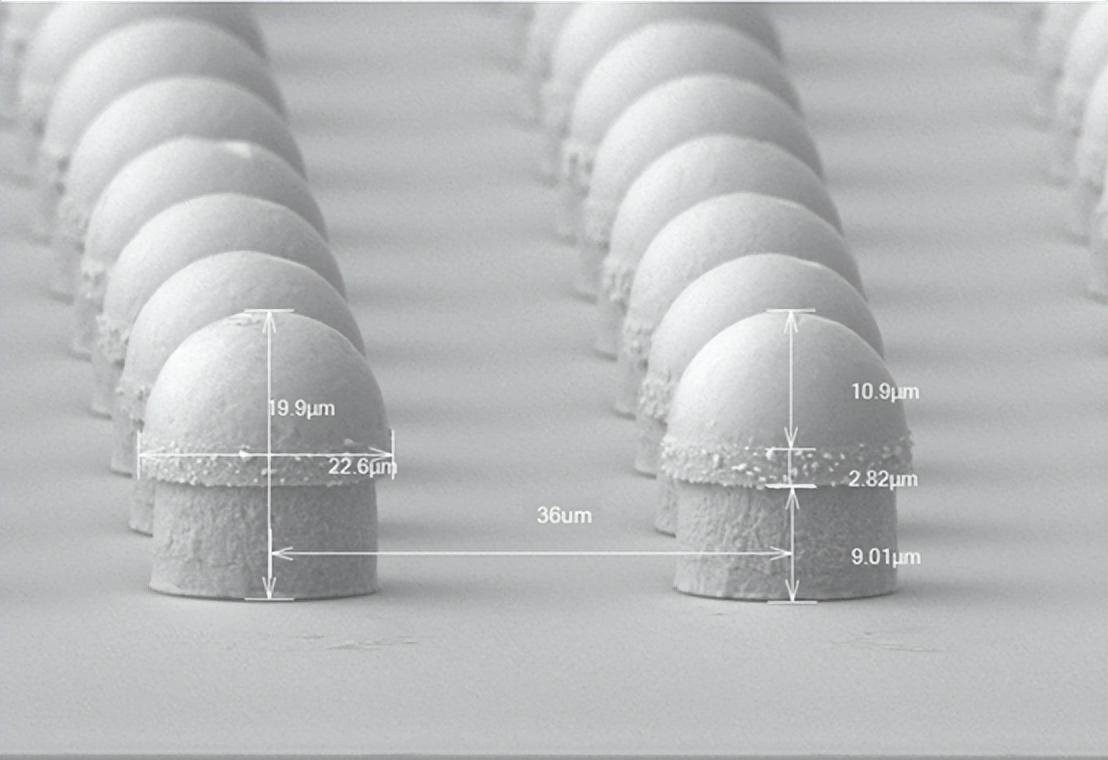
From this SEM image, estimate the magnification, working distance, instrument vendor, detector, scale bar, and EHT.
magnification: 1.46 K X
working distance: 22 mm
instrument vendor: Hitachi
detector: LA5(UL)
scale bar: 40000 nm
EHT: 15 kV